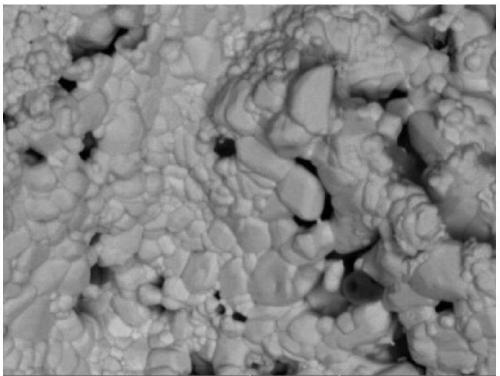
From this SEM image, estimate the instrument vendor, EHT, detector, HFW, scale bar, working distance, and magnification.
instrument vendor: Tescan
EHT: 20 kV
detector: BSE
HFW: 55.4 µm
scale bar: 10000 nm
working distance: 15.03 mm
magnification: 5 K X